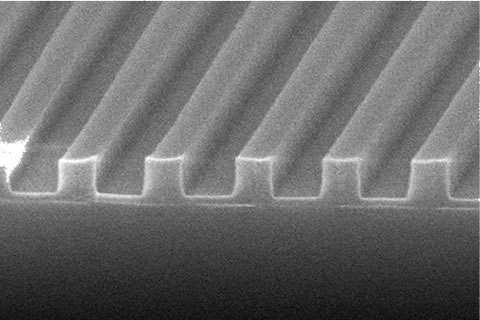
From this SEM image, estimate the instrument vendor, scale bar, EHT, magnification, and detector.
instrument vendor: JEOL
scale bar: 500 nm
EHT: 15 kV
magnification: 35 K X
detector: SEI